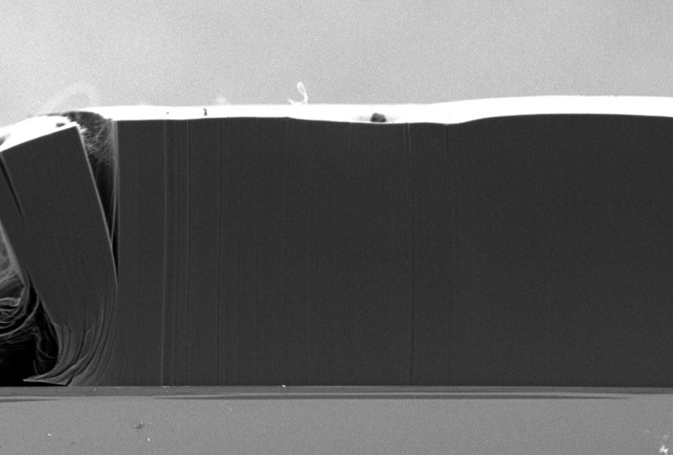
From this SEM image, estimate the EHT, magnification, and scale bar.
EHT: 2 kV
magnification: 0.1 K X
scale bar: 100000 nm